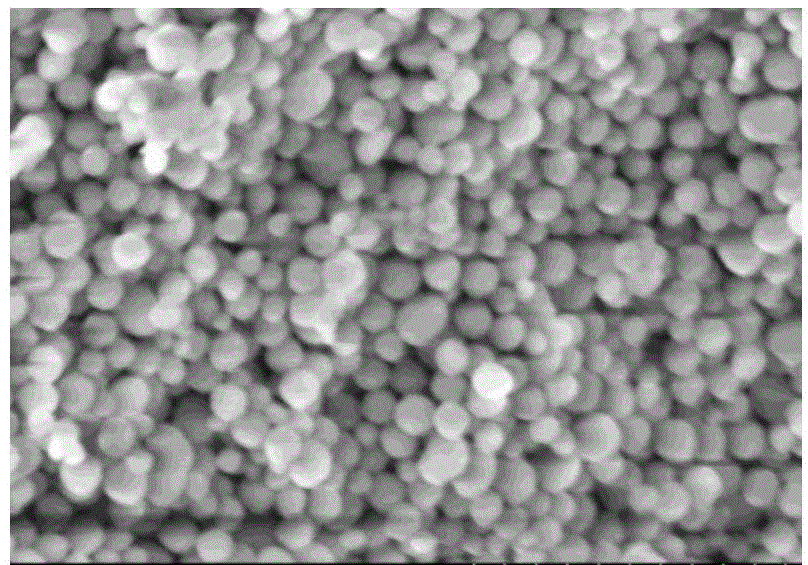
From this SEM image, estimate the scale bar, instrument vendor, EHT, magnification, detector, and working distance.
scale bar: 1000 nm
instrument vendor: Hitachi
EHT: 3 kV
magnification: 50 K X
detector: SE(M)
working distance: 9.2 mm